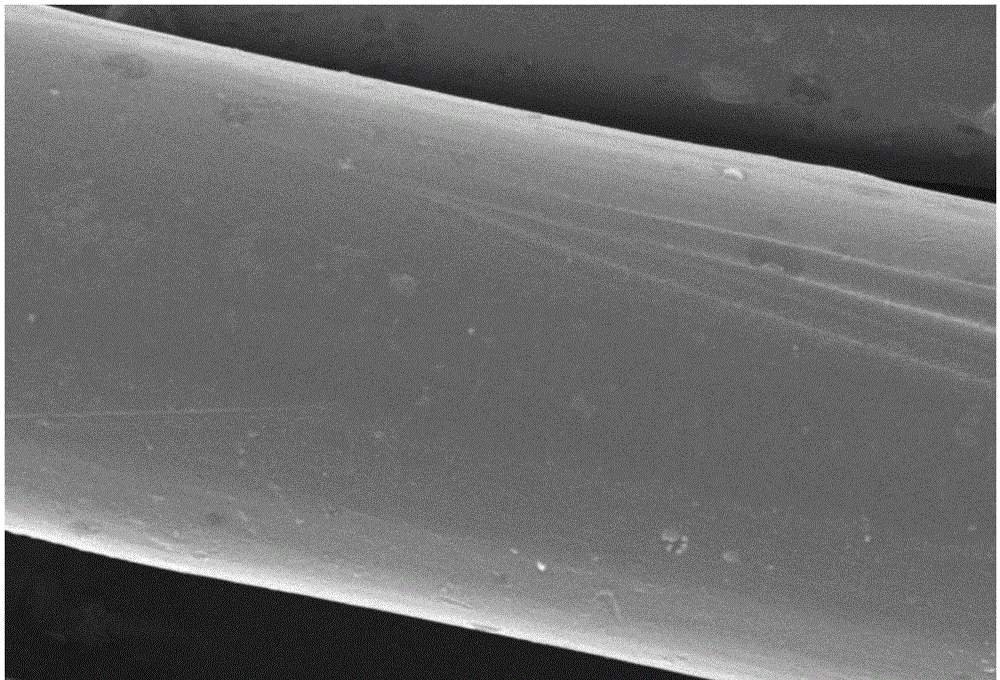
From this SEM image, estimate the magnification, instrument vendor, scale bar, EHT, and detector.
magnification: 1 K X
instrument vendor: JEOL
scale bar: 10000 nm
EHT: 15 kV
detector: SEI